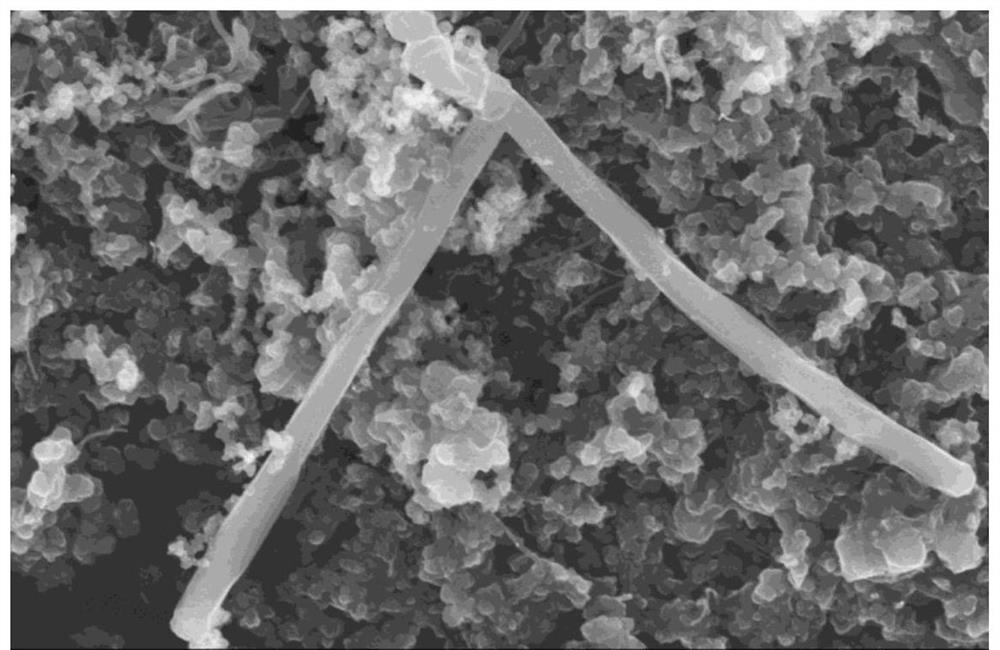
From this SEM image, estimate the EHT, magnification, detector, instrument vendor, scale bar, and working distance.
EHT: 5 kV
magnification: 25 K X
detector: SE(M)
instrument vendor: Hitachi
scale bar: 2000 nm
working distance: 6.3 mm